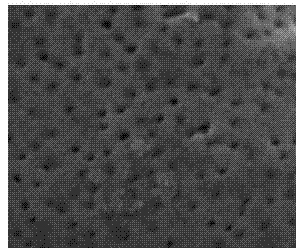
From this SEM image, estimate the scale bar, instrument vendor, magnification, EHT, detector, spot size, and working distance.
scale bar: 500 nm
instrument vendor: FEI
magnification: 120 K X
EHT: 10 kV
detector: TLD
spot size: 3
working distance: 5.1 mm